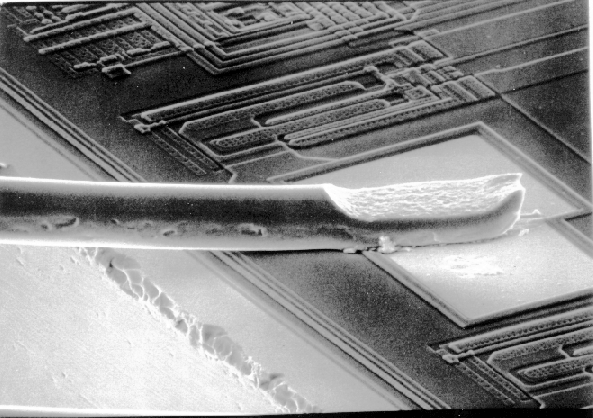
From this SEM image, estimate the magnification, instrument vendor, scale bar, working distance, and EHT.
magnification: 0.4 K X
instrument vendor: JEOL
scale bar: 10000 nm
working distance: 53 mm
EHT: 20 kV